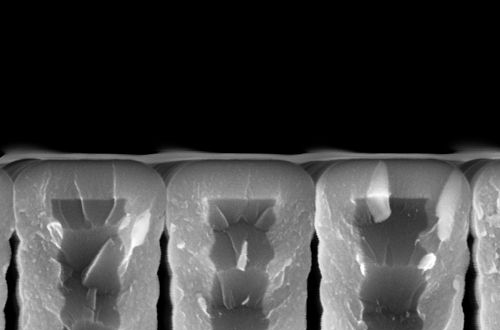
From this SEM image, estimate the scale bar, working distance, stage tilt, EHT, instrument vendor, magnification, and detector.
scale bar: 200 nm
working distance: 3.4 mm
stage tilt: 0°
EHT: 5 kV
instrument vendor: Zeiss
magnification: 229.84 K X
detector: InLens